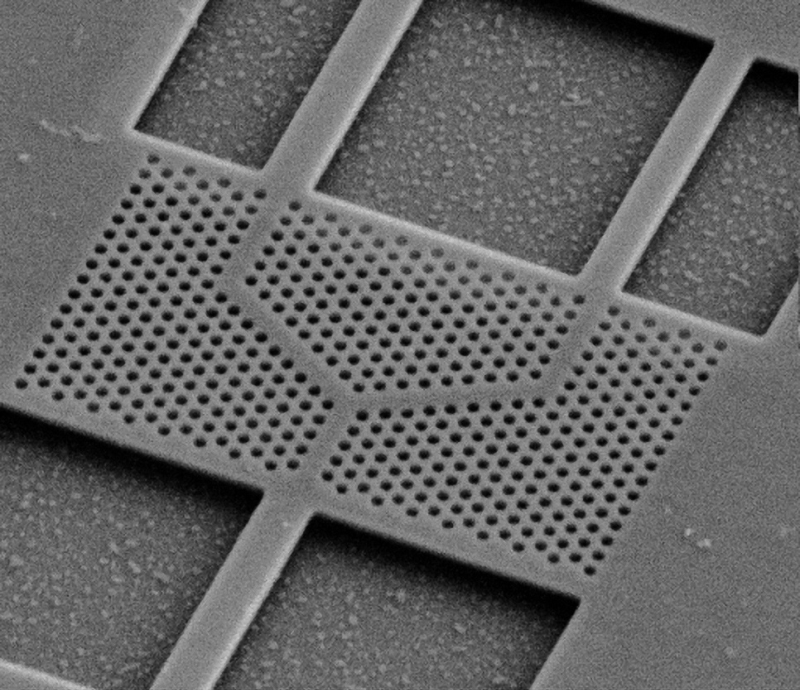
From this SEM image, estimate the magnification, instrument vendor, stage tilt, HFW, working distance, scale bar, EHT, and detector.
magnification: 10 K X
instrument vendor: FEI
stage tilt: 45°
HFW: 15.2 µm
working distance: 5.099 mm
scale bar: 2000 nm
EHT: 5 kV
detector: SED